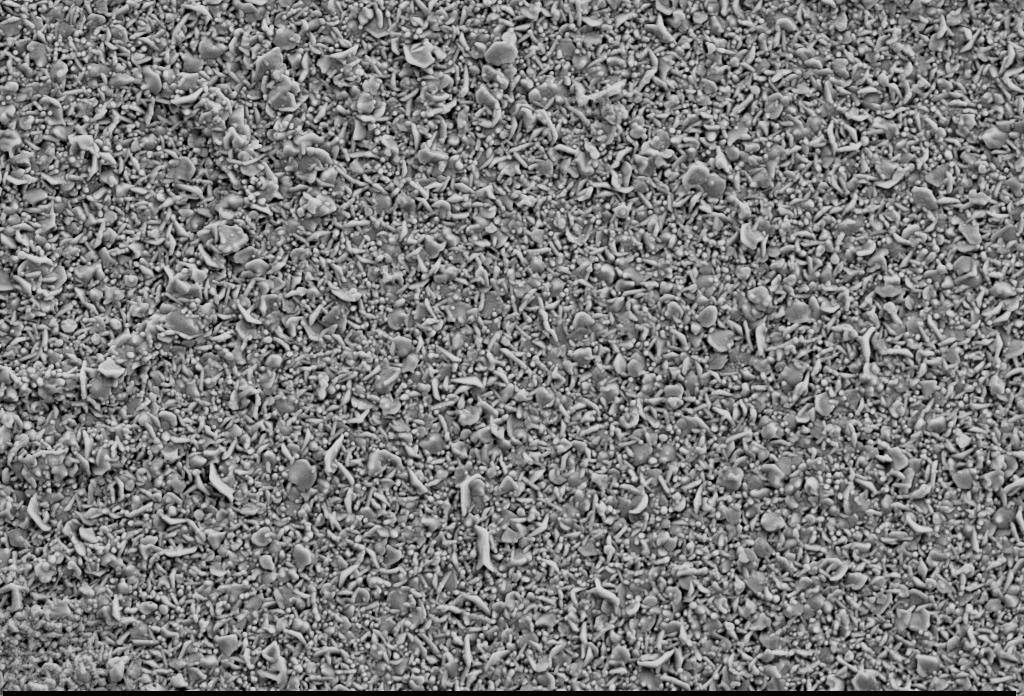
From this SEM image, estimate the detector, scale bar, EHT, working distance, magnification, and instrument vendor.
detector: SE2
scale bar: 2000 nm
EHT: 5 kV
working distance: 8.1 mm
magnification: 20 K X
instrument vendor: Zeiss